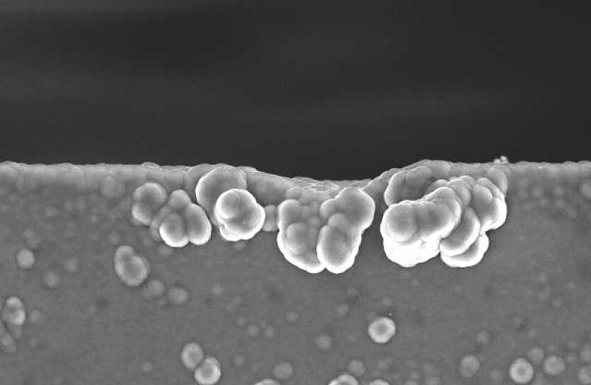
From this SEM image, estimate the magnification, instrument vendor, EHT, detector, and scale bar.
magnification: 1 K X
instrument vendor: JEOL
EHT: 20 kV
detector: SEI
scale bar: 10000 nm